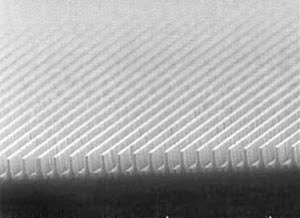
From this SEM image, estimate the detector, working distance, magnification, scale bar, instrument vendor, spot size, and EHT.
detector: SE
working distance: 4.9 mm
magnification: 18.826 K X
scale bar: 2000 nm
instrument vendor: FEI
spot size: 3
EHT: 10 kV